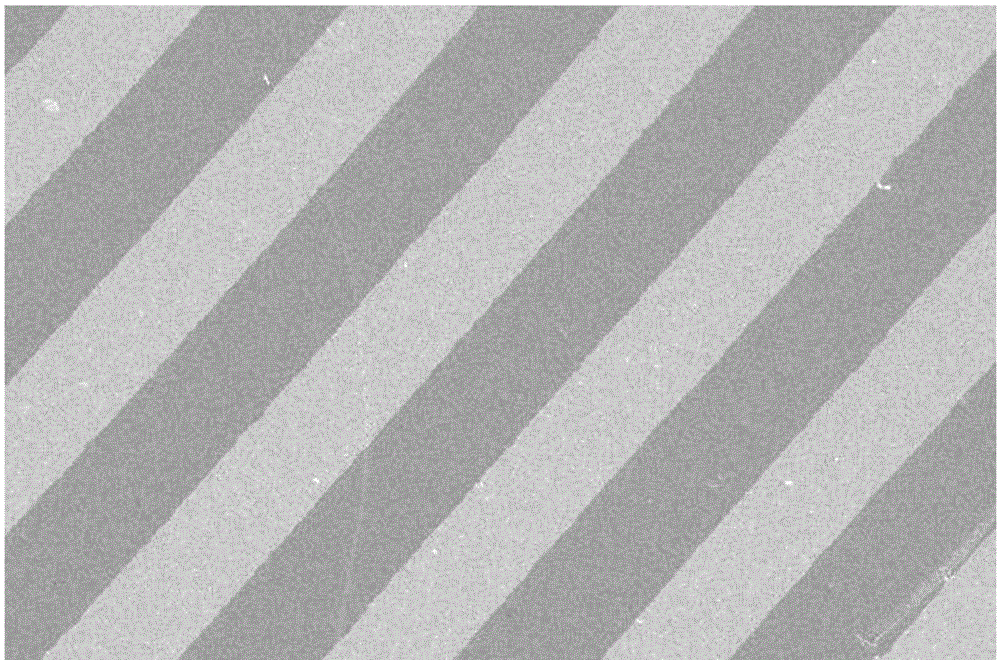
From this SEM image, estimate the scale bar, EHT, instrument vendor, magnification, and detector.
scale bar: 100000 nm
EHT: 15 kV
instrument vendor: JEOL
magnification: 0.17 K X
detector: SEI